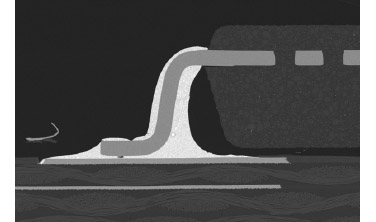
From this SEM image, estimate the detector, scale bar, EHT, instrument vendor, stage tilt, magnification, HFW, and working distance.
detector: T1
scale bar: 1000000 nm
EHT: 30 kV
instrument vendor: FEI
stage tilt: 0°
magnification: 0.15 K X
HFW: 2760 µm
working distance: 51.4 mm